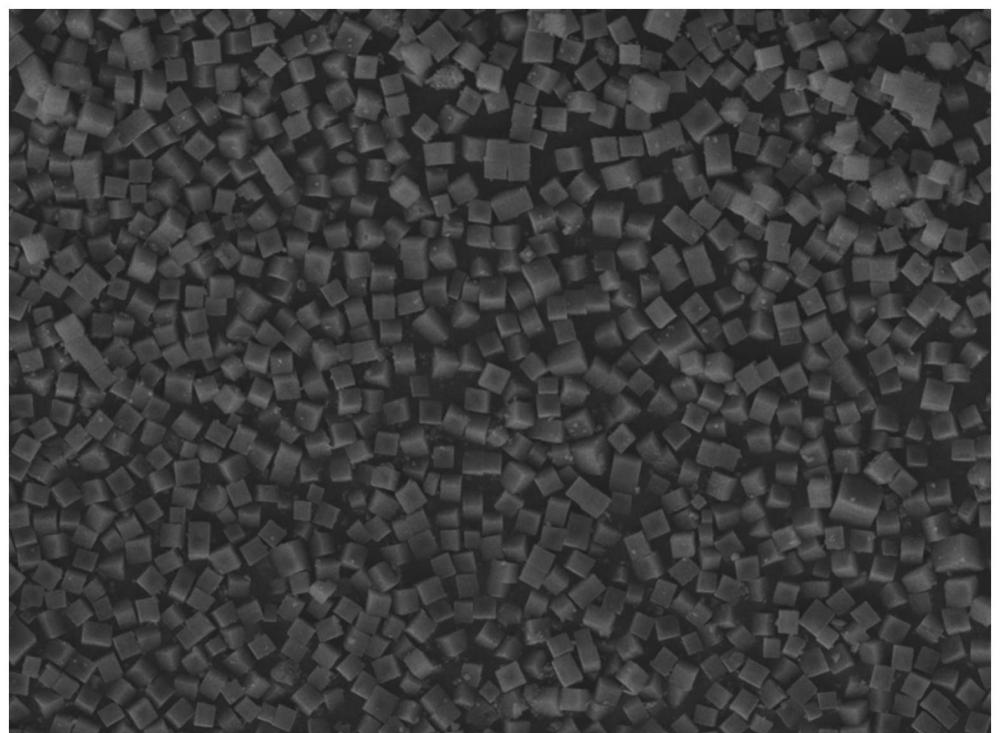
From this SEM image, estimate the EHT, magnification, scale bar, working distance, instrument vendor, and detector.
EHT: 15 kV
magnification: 0.95 K X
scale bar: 20000 nm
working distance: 10 mm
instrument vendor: JEOL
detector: SED